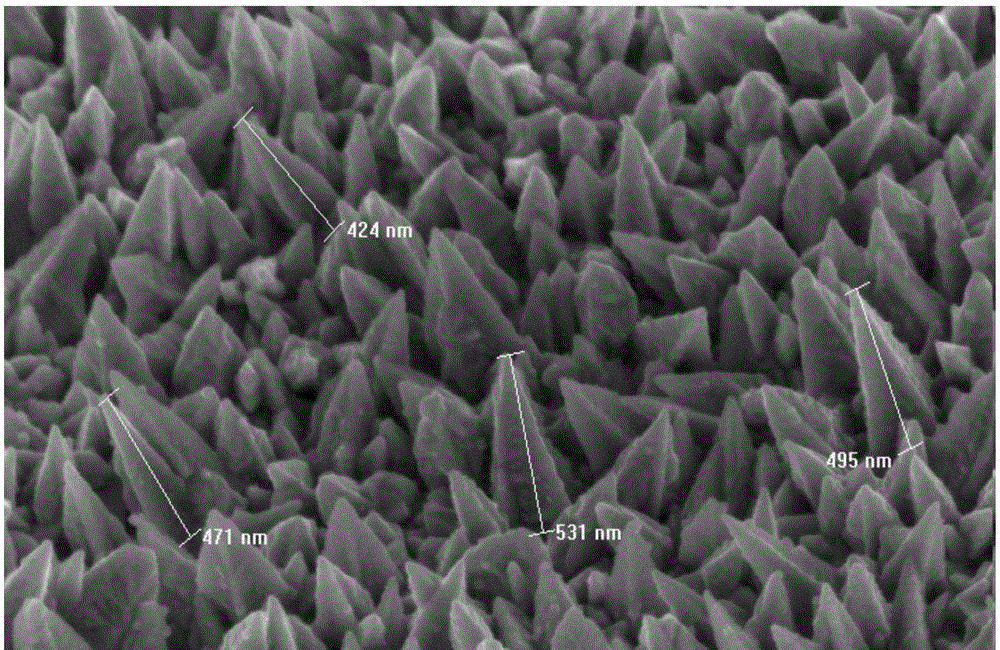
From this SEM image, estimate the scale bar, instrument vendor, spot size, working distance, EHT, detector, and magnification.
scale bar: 500 nm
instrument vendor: FEI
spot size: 4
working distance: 5.5 mm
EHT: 5 kV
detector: TLD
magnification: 100 K X